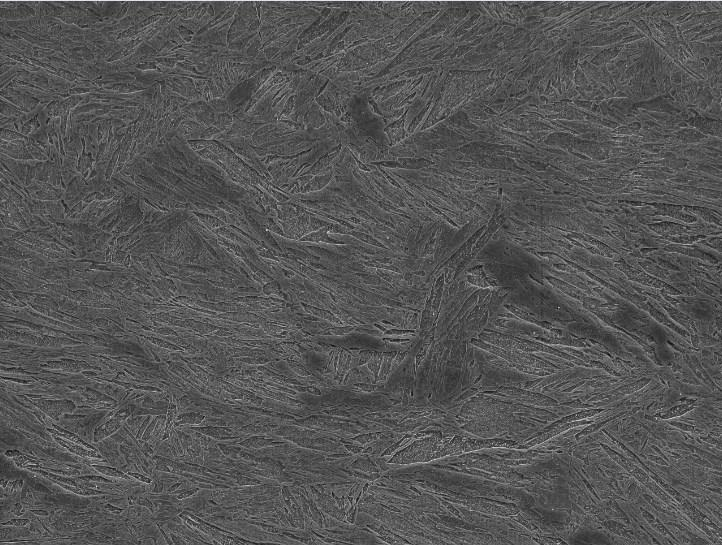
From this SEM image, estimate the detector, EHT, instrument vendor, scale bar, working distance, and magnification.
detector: SEI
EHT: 15 kV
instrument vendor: JEOL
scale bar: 10000 nm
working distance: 9.8 mm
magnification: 2.2 K X